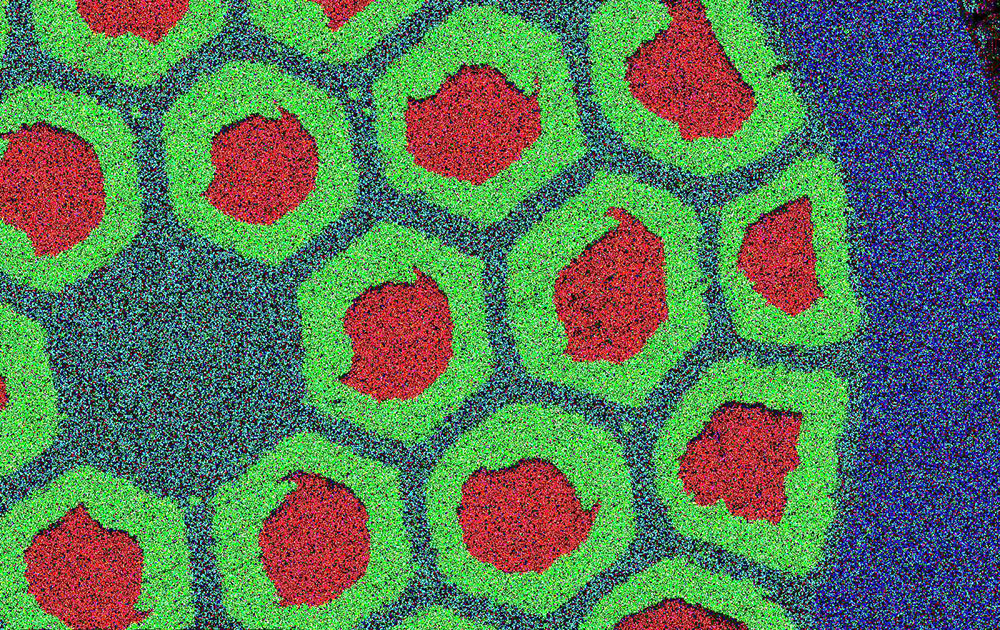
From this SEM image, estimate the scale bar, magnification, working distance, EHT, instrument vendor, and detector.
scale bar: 70000 nm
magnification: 0.5 K X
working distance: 10 mm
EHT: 15 kV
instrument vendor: other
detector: SE